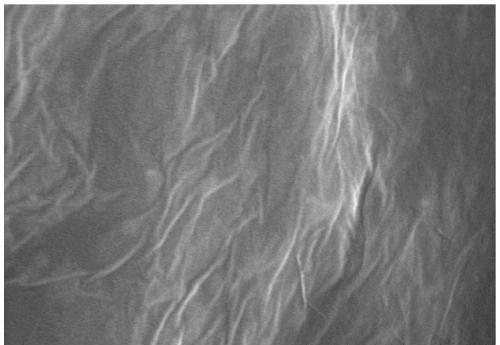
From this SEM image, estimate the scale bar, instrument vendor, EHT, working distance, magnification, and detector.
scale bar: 500 nm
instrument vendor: Hitachi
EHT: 10 kV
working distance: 9.6 mm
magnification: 100 K X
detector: SE(M)